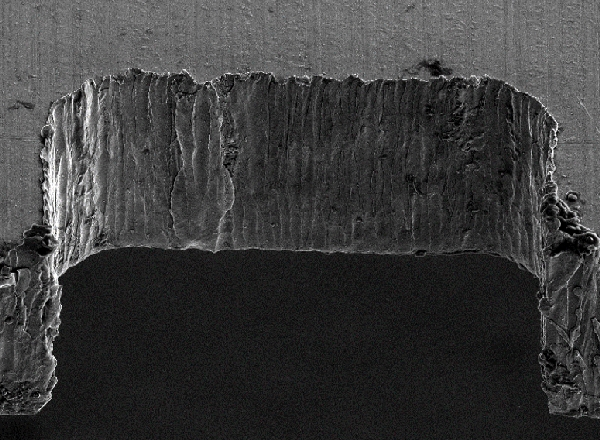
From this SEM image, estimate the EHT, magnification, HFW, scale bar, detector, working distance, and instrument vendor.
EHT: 10 kV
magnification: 0.35 K X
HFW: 363 µm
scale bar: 100000 nm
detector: ETD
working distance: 7.8 mm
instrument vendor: FEI